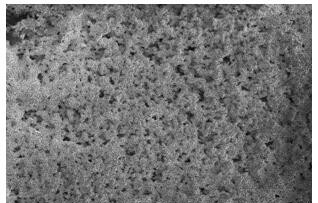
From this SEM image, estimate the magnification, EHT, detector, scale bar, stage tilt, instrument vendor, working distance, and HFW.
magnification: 0.2 K X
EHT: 2 kV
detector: ETD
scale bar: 200000 nm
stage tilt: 0°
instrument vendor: FEI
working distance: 5.9 mm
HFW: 625 µm